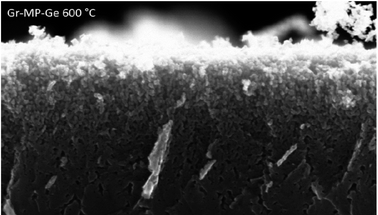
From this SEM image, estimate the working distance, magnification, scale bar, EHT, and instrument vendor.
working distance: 2.8 mm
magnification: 50 K X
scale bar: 100 nm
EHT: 20 kV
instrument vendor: Zeiss